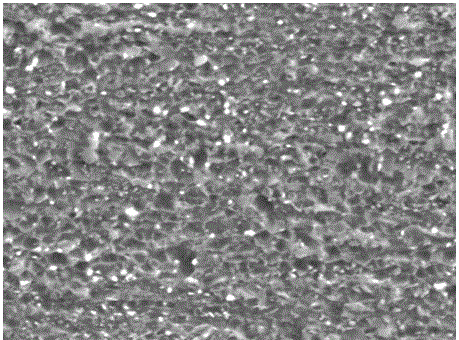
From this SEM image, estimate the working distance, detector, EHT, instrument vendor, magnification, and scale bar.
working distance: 10.9 mm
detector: SEI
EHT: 20 kV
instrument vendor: JEOL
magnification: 6 K X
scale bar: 1000 nm